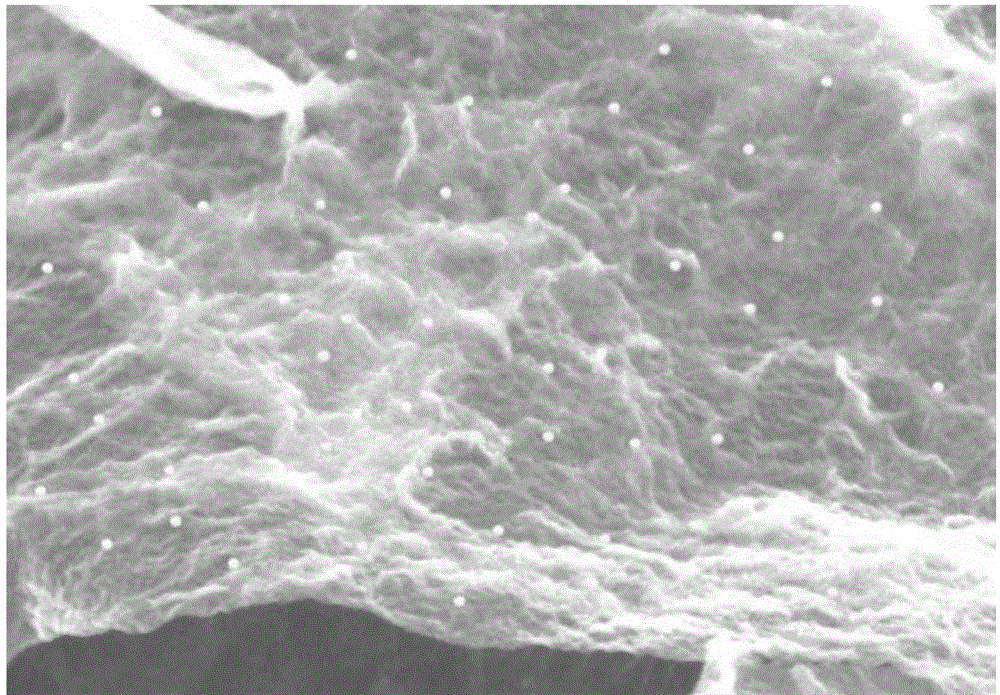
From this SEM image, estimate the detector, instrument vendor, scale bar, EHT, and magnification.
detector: SE(M)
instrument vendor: Hitachi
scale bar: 1000 nm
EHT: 4 kV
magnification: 40 K X